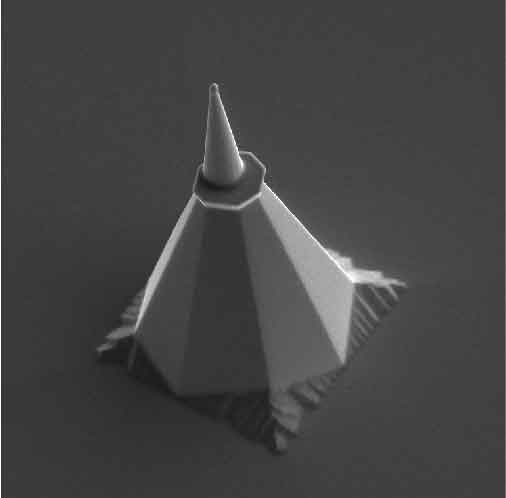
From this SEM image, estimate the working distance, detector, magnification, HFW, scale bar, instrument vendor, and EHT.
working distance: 8.86 mm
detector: SE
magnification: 1.51 K X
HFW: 137 µm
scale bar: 20000 nm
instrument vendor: Tescan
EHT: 10 kV